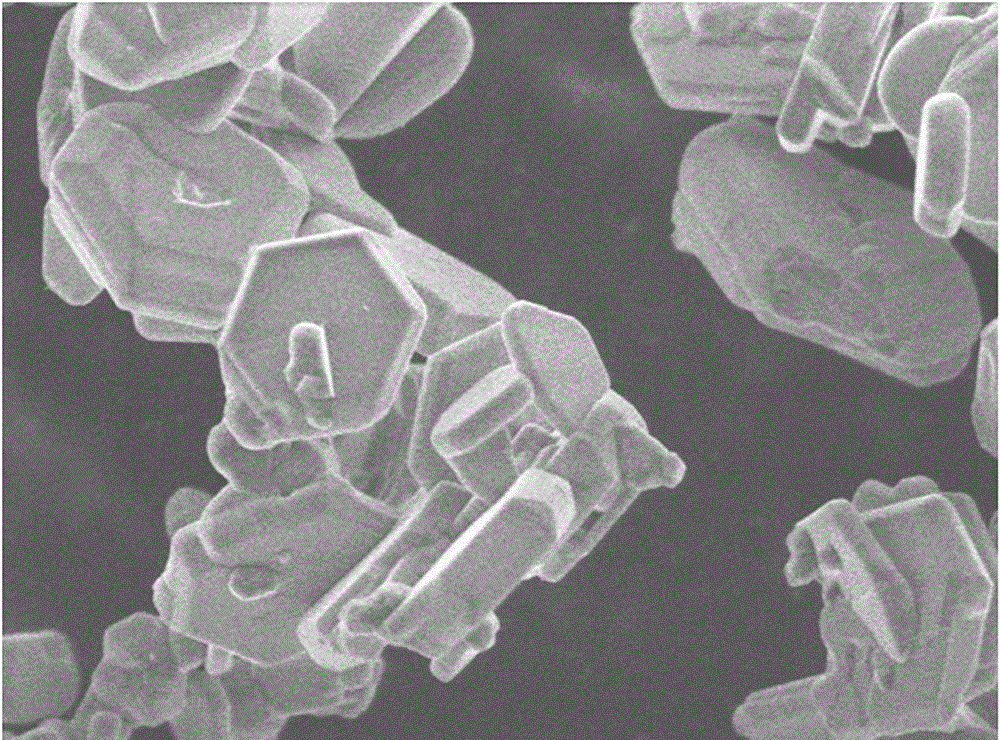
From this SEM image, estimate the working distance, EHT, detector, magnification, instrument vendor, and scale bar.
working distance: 6.6 mm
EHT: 5 kV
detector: SE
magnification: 5 K X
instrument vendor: Hitachi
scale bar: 10000 nm